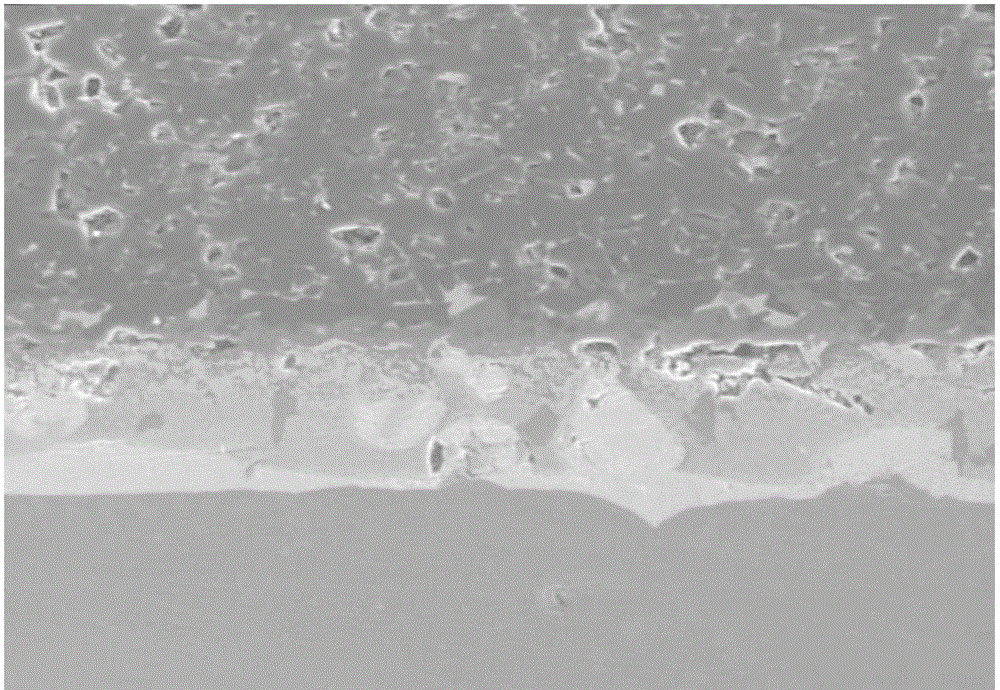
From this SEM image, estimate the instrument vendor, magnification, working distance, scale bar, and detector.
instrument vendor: Hitachi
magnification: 1 K X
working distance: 13.6 mm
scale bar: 50000 nm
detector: SE(M)